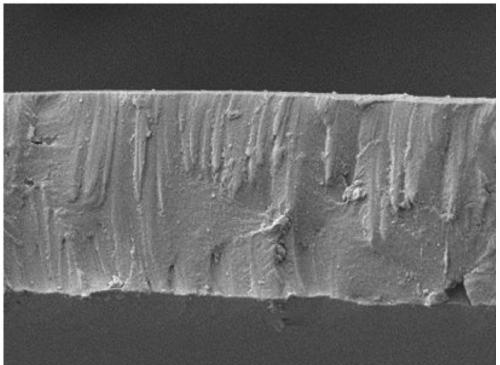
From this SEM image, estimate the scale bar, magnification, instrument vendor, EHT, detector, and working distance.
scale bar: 10000 nm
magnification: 2 K X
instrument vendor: JEOL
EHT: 5 kV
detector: SEM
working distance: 11.5 mm